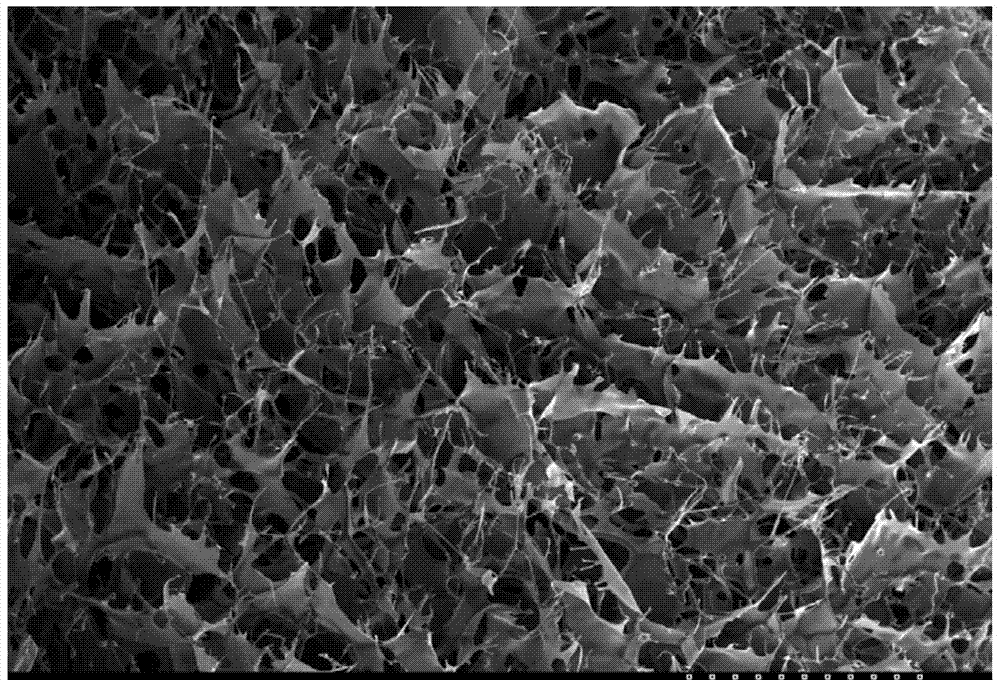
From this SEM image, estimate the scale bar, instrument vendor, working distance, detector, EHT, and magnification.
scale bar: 1000000 nm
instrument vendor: Hitachi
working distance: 23.1 mm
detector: SE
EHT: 20 kV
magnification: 0.03 K X